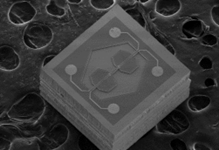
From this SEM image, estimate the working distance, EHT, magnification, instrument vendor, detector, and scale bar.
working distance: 8 mm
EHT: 3 kV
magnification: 0.07 K X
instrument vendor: Hitachi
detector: SE(M)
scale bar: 500000 nm